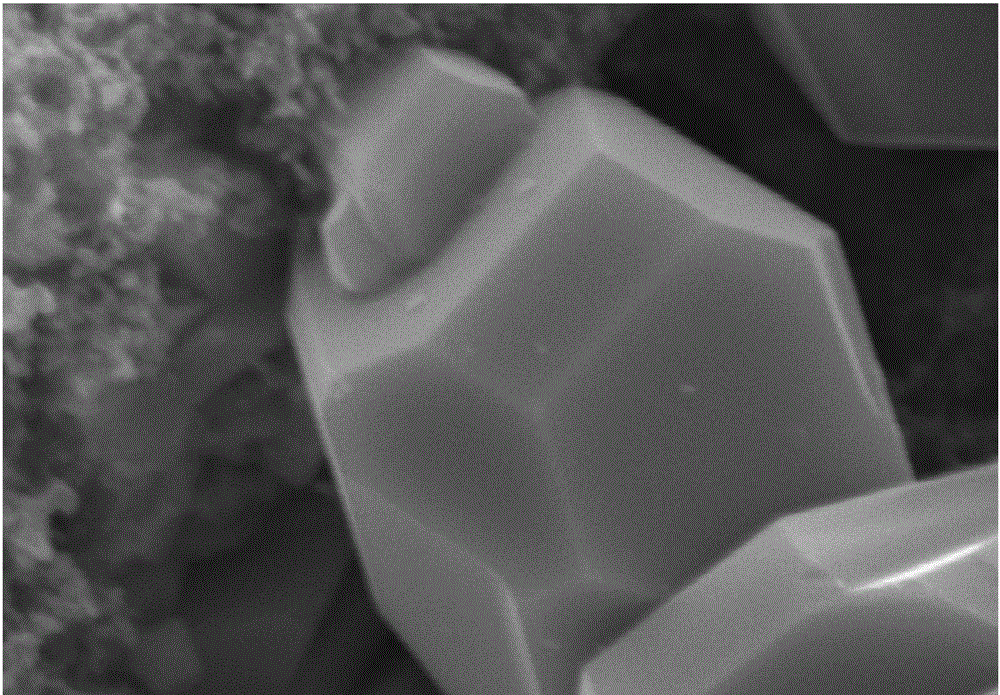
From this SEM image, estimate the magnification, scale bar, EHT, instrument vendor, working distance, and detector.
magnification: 15 K X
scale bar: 3000 nm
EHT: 15 kV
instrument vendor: Hitachi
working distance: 10 mm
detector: SE